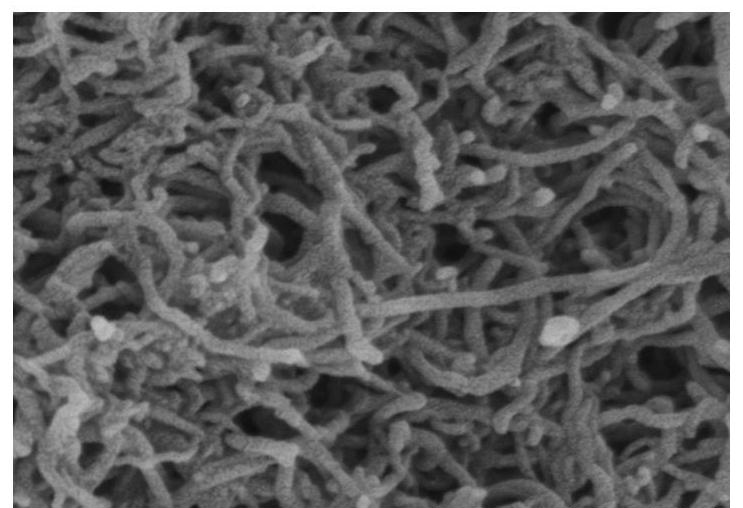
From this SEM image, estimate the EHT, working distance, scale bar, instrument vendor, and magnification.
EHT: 3 kV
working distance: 7.9 mm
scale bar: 500 nm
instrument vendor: Hitachi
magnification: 100 K X